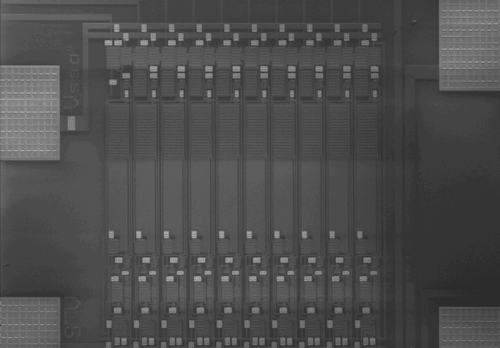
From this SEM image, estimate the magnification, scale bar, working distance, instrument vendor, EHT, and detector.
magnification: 0.04 K X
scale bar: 1000000 nm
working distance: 8.3 mm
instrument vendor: Hitachi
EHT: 1 kV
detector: LM(UL)